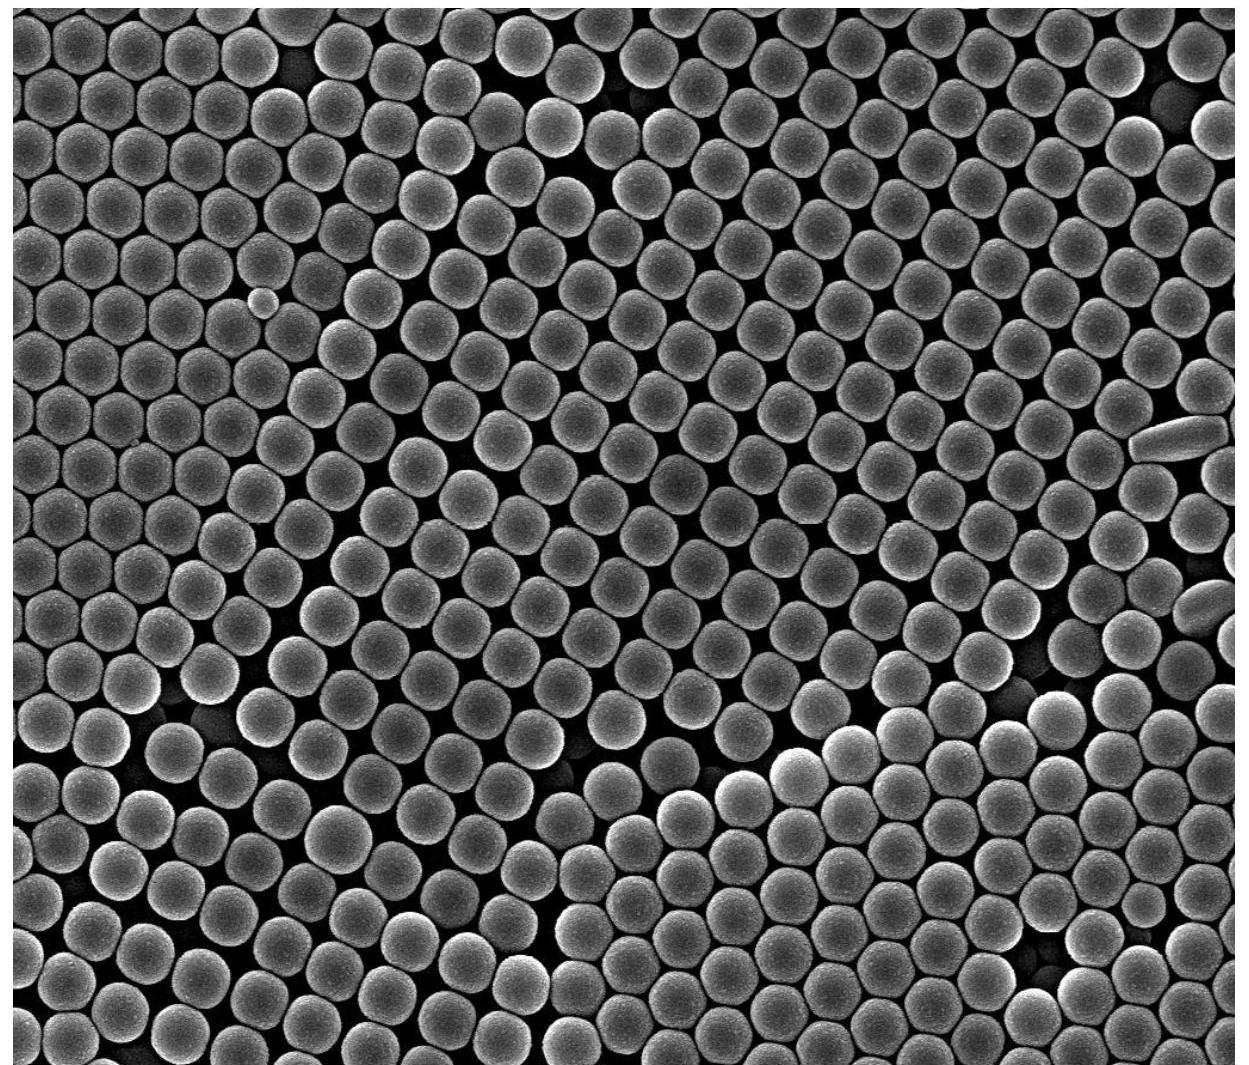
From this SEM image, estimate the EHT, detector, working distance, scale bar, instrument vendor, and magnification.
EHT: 20 kV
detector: SE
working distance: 10.2 mm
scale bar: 3000 nm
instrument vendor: FEI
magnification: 40 K X